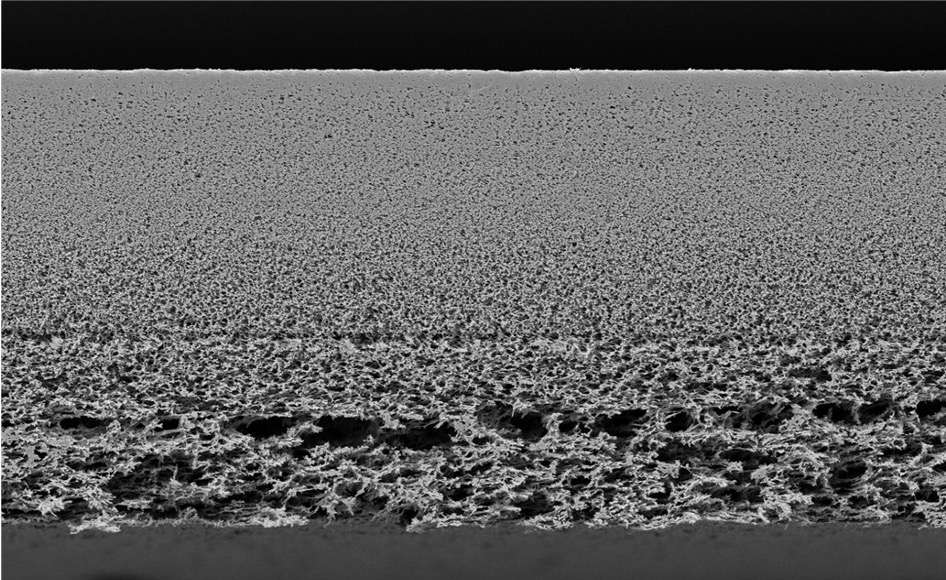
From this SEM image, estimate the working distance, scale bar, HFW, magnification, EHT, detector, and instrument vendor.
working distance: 5.678 mm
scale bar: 100000 nm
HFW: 318 µm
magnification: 0.4 K X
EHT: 10 kV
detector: Mix 50%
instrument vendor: other